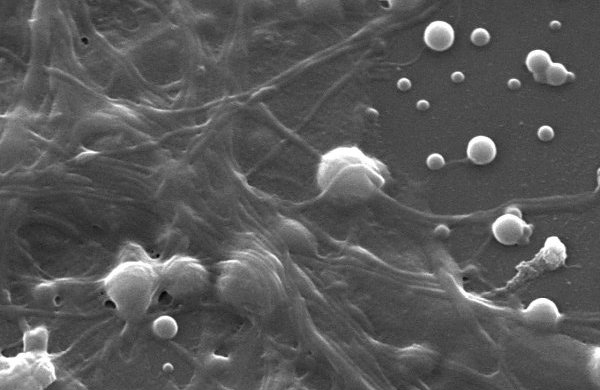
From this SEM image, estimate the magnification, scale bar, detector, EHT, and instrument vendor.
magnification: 8.5 K X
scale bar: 2000 nm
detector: SEI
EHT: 15 kV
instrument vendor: JEOL